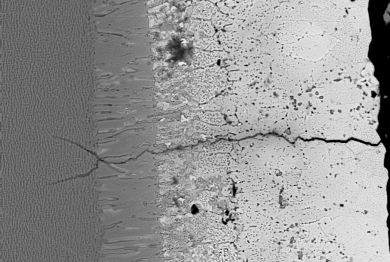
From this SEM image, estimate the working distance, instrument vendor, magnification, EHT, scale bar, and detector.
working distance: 19 mm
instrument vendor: Zeiss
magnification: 1 K X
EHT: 15 kV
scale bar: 10000 nm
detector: QBSD